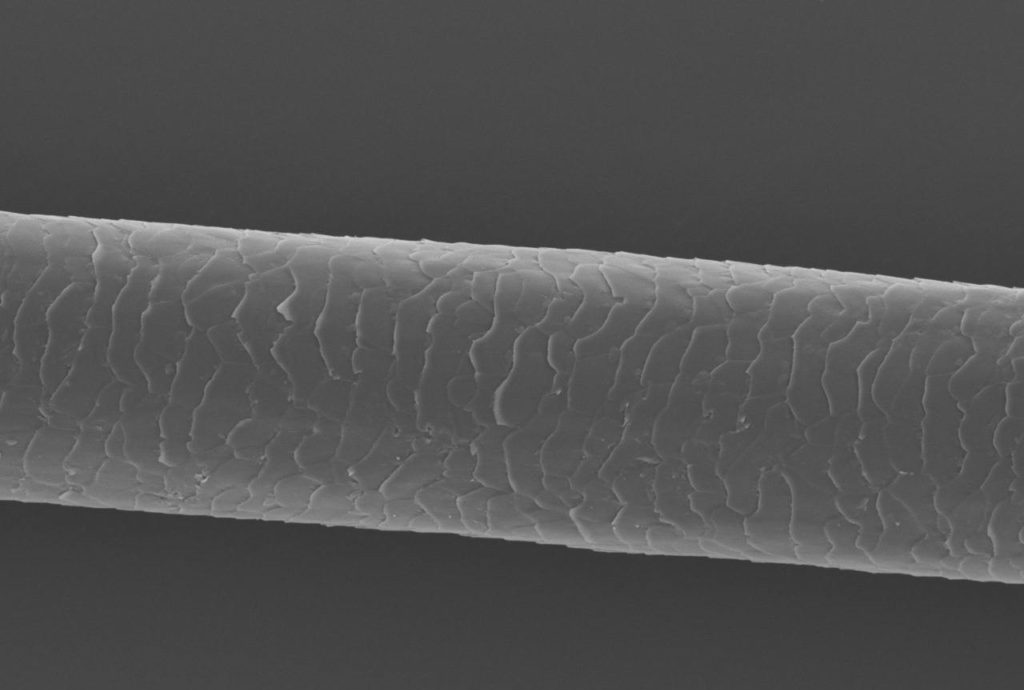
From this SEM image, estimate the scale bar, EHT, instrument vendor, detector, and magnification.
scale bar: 50000 nm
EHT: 10 kV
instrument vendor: JEOL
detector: SEI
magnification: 0.5 K X